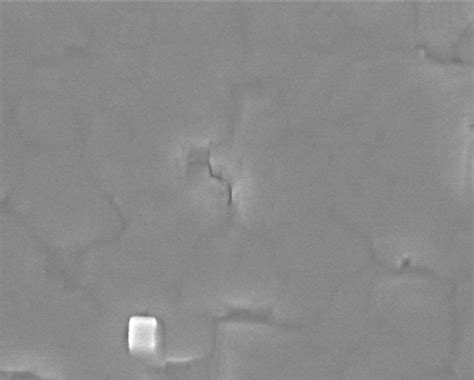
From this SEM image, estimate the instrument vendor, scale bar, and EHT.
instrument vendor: Hitachi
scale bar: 500 nm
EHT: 5 kV